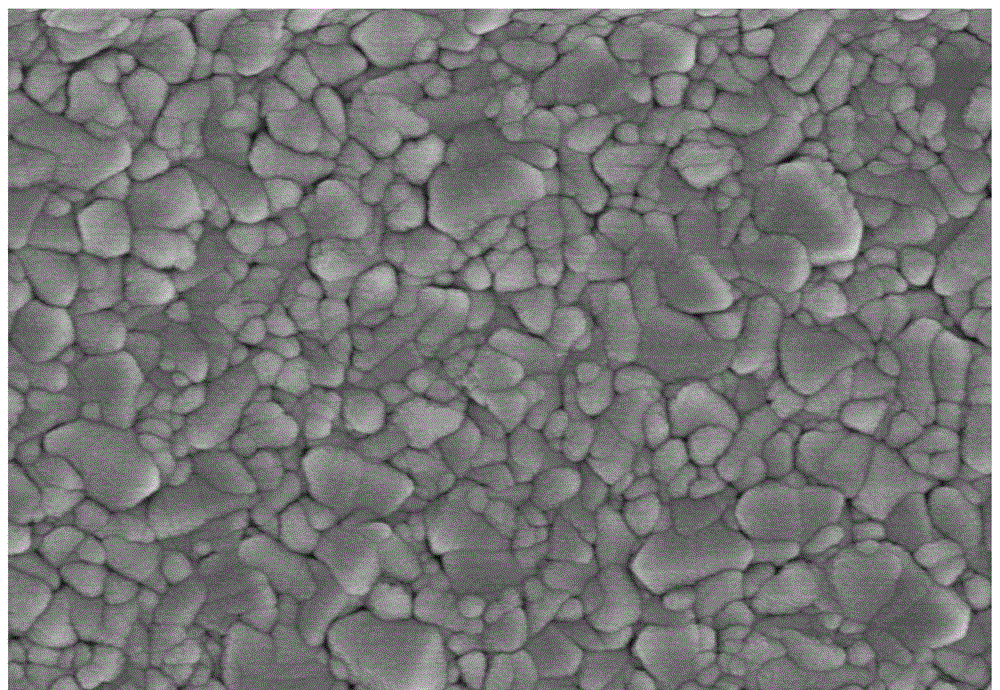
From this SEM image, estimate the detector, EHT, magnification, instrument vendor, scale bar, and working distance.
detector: SE(UL)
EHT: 5 kV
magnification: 30 K X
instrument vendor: Hitachi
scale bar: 1000 nm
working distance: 8.5 mm